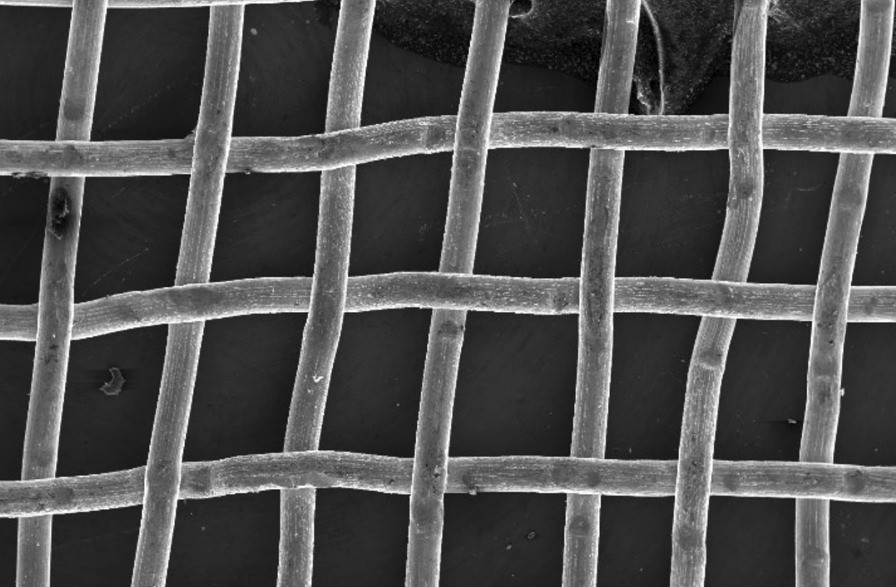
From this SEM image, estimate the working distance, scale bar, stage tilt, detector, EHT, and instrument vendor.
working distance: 14.5 mm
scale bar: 1000000 nm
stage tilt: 0°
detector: SE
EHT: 20 kV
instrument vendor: FEI